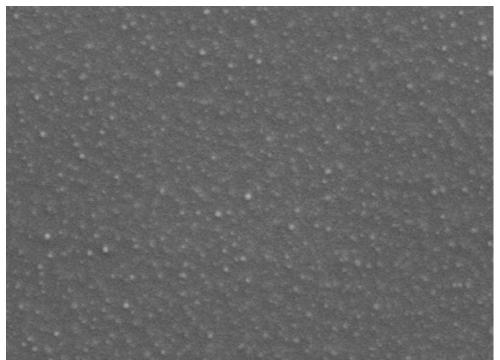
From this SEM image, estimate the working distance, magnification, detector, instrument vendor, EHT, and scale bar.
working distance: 8.2 mm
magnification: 30 K X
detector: LED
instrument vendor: JEOL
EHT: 1 kV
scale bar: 100 nm